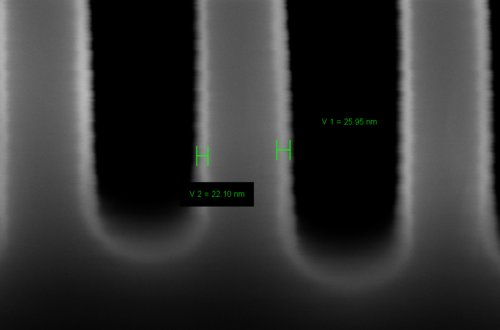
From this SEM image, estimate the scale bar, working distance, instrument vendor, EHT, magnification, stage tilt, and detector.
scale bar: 100 nm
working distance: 4.1 mm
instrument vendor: Zeiss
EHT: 5 kV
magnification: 305.89 K X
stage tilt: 0°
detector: InLens